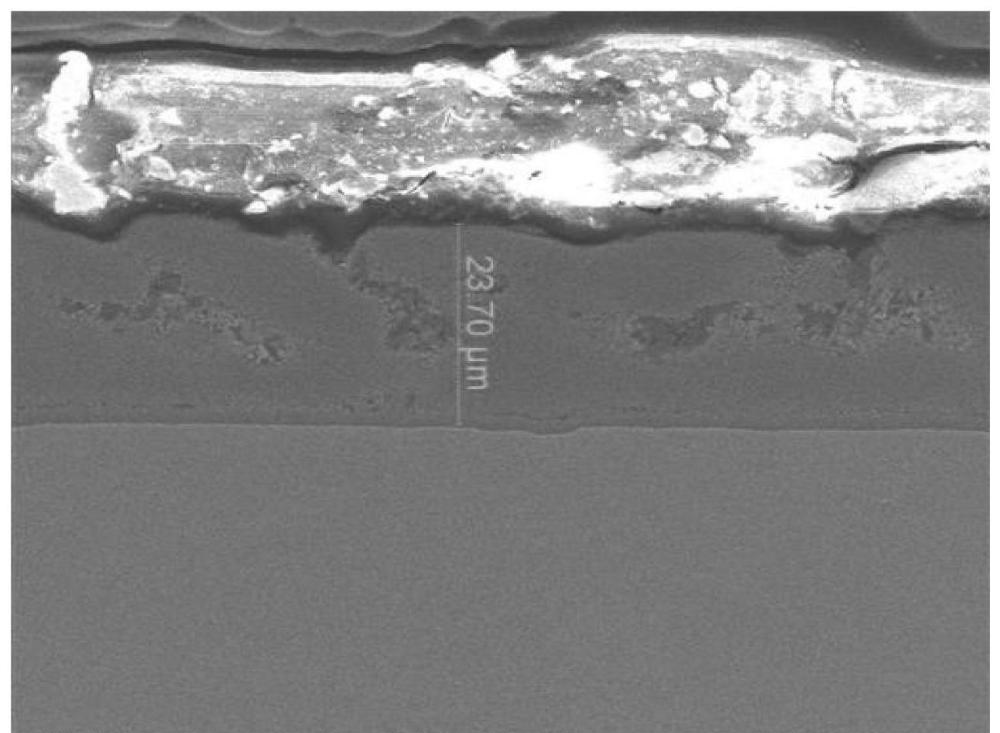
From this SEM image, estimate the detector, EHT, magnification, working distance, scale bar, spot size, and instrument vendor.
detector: ETD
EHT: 15 kV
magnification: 1.5 K X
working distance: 9.9 mm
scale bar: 40000 nm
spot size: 3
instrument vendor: FEI